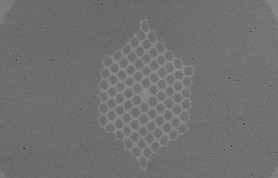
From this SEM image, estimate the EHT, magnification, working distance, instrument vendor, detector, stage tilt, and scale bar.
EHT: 5 kV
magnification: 2.43 K X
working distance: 4.5 mm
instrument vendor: Zeiss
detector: SE2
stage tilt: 0°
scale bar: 2000 nm